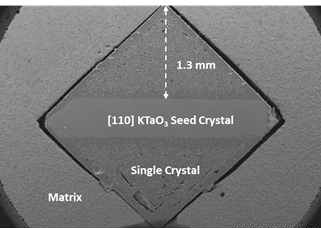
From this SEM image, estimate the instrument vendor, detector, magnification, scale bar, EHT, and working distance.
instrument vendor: Hitachi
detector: SE(U)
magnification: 0.03 K X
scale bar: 1000000 nm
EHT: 15 kV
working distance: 11.9 mm